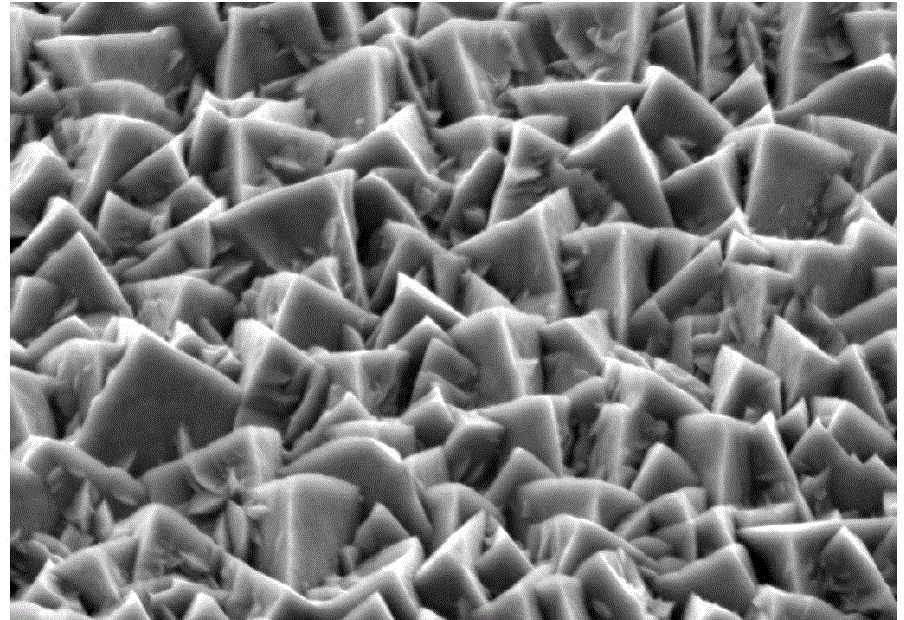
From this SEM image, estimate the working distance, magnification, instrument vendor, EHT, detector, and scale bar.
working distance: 5.5 mm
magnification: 20 K X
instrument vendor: Zeiss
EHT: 5 kV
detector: SE2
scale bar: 200 nm